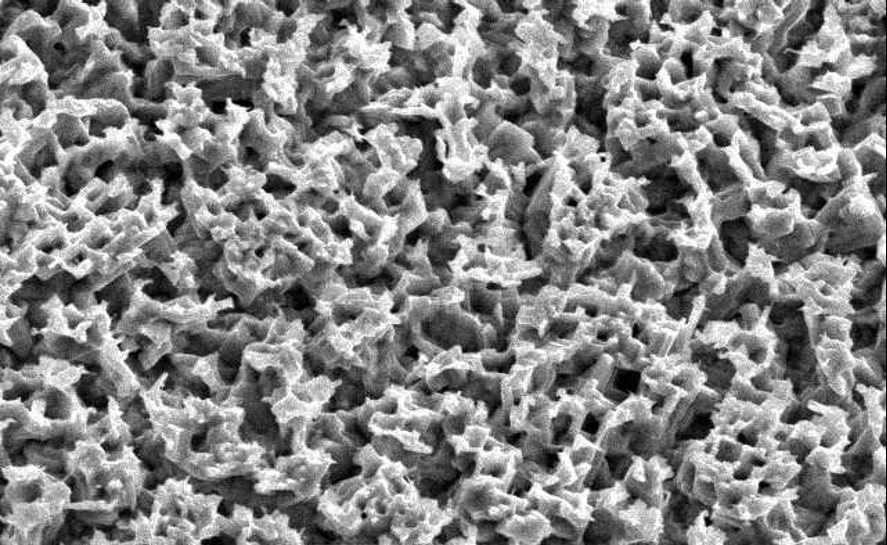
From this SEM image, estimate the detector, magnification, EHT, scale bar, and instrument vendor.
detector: SEI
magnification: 5 K X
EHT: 15 kV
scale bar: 5000 nm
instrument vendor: JEOL